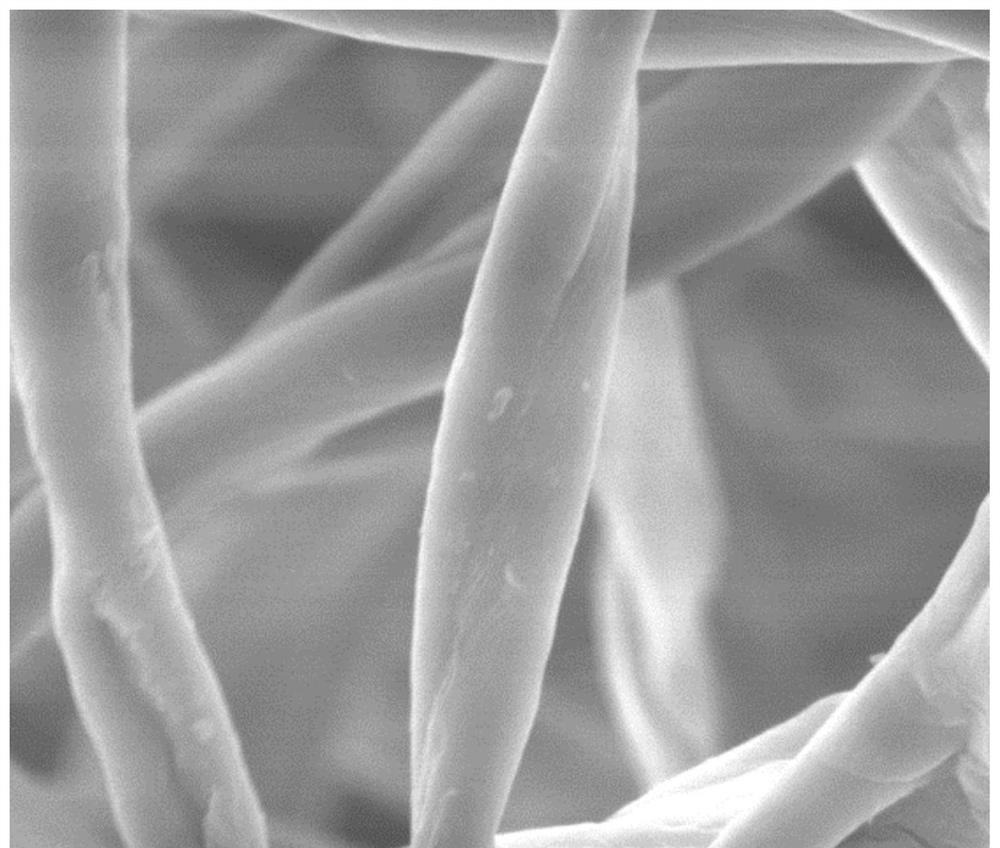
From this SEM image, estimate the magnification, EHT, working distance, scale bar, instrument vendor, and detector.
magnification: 3 K X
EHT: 20 kV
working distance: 12.9 mm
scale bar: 20000 nm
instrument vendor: FEI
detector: ETD